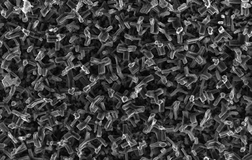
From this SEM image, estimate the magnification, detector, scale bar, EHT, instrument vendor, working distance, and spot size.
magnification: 5 K X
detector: SE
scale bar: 5000 nm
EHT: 5 kV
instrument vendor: FEI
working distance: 13.8 mm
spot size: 2